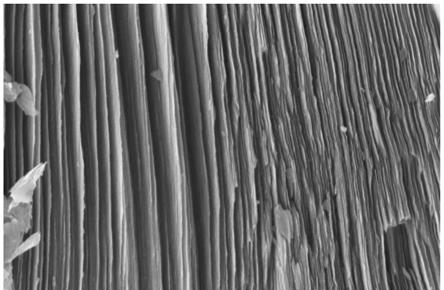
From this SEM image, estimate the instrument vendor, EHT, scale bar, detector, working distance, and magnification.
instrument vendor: Zeiss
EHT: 5 kV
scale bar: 200 nm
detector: InLens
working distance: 7.2 mm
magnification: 20 K X